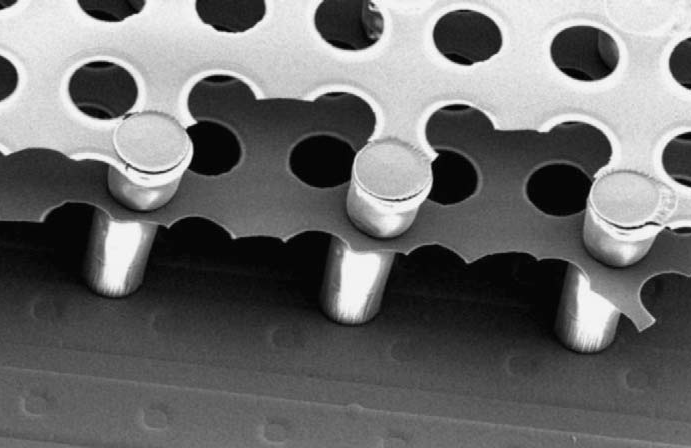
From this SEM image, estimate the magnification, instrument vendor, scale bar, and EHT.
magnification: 0.4 K X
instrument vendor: JEOL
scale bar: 50000 nm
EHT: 6 kV